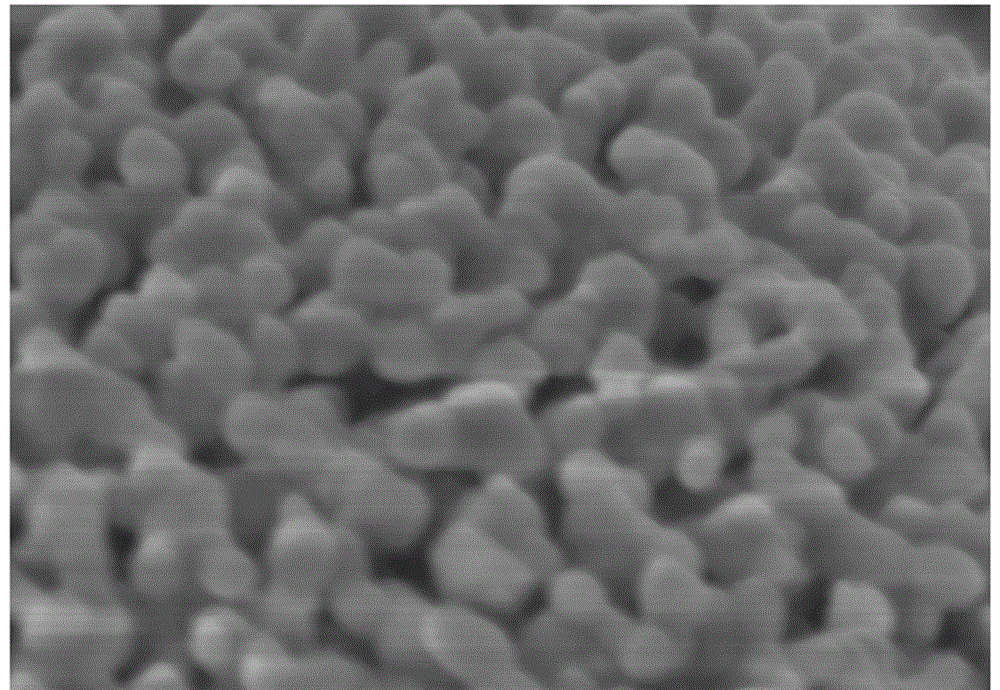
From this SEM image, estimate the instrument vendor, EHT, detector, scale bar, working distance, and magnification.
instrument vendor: Hitachi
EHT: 3 kV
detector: SE(M)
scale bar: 300 nm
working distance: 3.5 mm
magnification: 150 K X